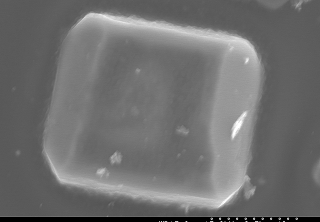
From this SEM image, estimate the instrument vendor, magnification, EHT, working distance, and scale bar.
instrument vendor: JEOL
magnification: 3.5 K X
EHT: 15 kV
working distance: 15 mm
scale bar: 10000 nm